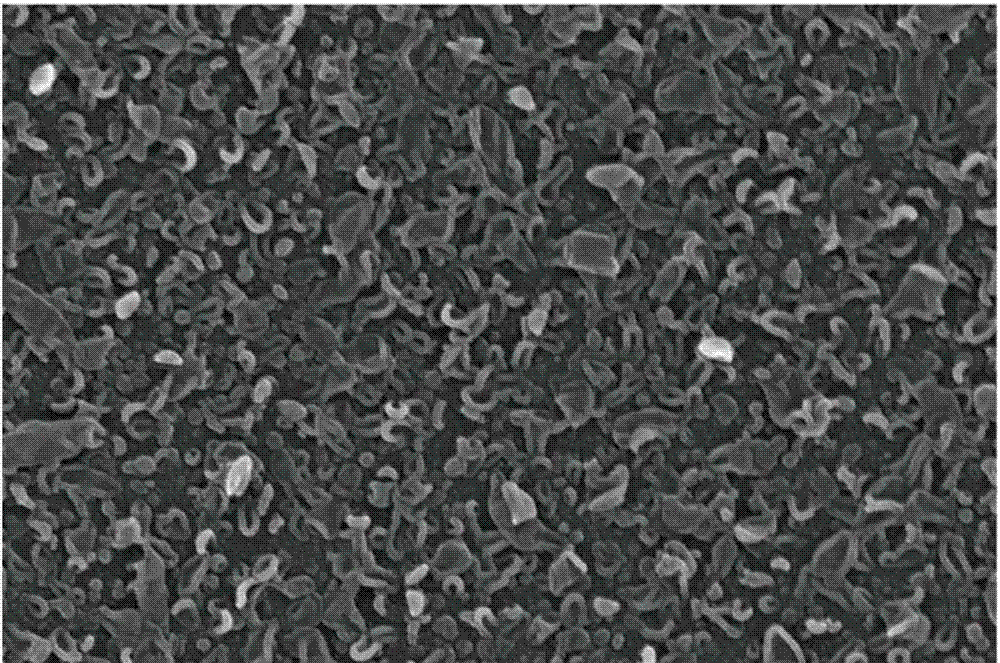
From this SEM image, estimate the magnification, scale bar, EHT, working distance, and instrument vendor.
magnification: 80 K X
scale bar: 2000 nm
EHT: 15 kV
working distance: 5.9 mm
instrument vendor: FEI